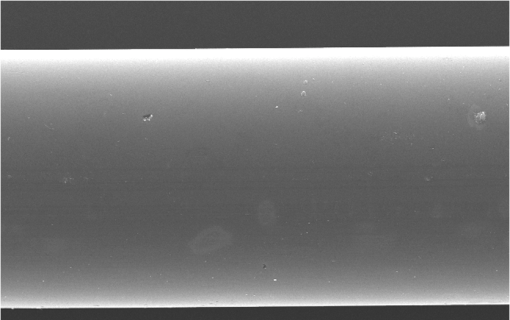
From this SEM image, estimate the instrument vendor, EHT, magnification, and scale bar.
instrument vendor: JEOL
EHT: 25 kV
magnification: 0.5 K X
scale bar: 50000 nm